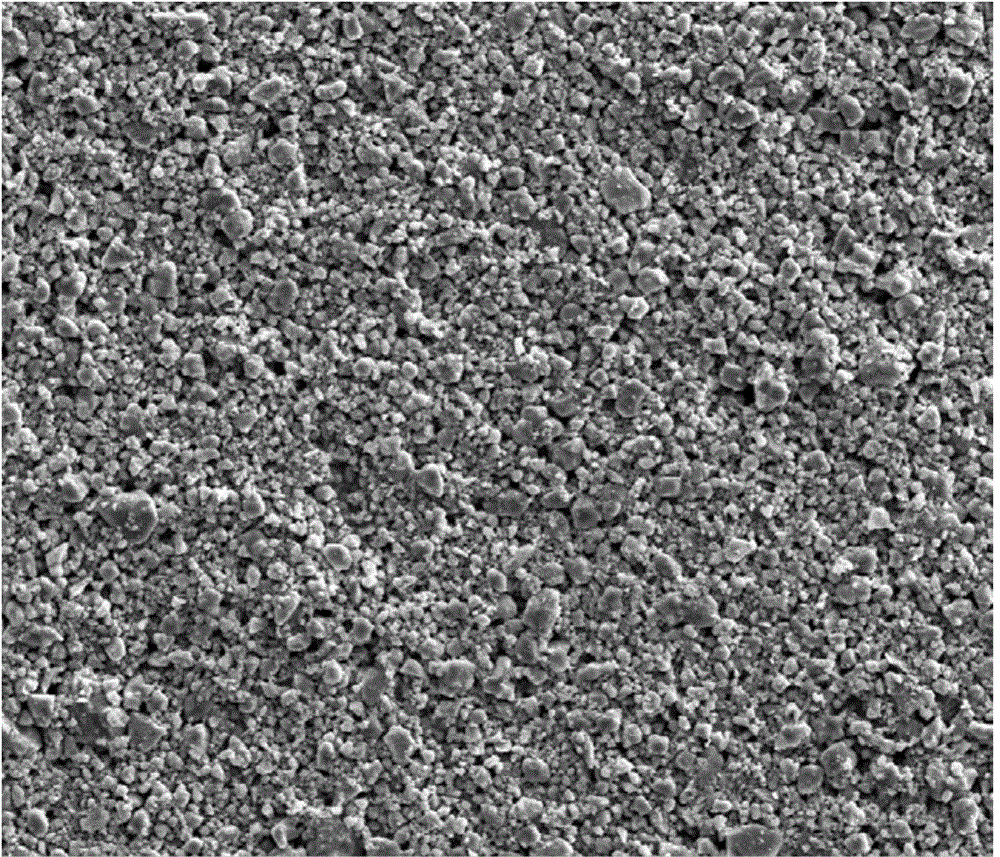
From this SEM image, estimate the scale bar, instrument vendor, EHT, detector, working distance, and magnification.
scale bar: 50000 nm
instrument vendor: FEI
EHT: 20 kV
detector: SE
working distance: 8.4 mm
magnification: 1 K X